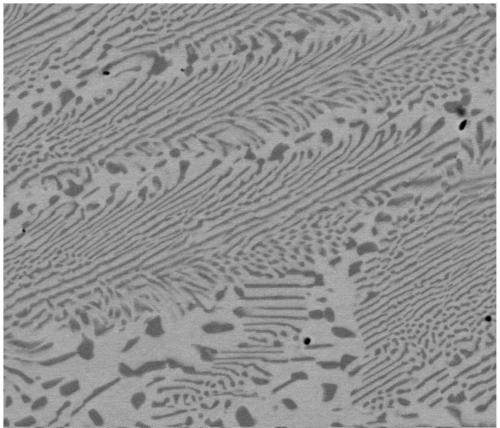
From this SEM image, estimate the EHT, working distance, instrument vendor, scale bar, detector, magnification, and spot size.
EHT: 20 kV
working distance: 13.9 mm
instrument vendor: FEI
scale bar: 20000 nm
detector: vCD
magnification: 5 K X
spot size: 5